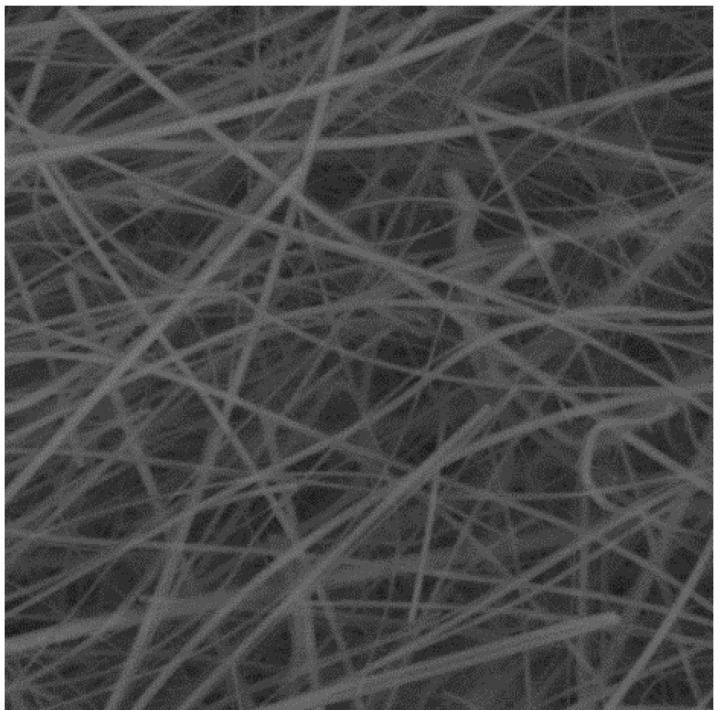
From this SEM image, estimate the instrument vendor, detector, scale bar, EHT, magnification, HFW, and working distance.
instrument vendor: Tescan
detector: BSE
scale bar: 20000 nm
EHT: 20 kV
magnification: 2 K X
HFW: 72.3 µm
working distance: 9.55 mm